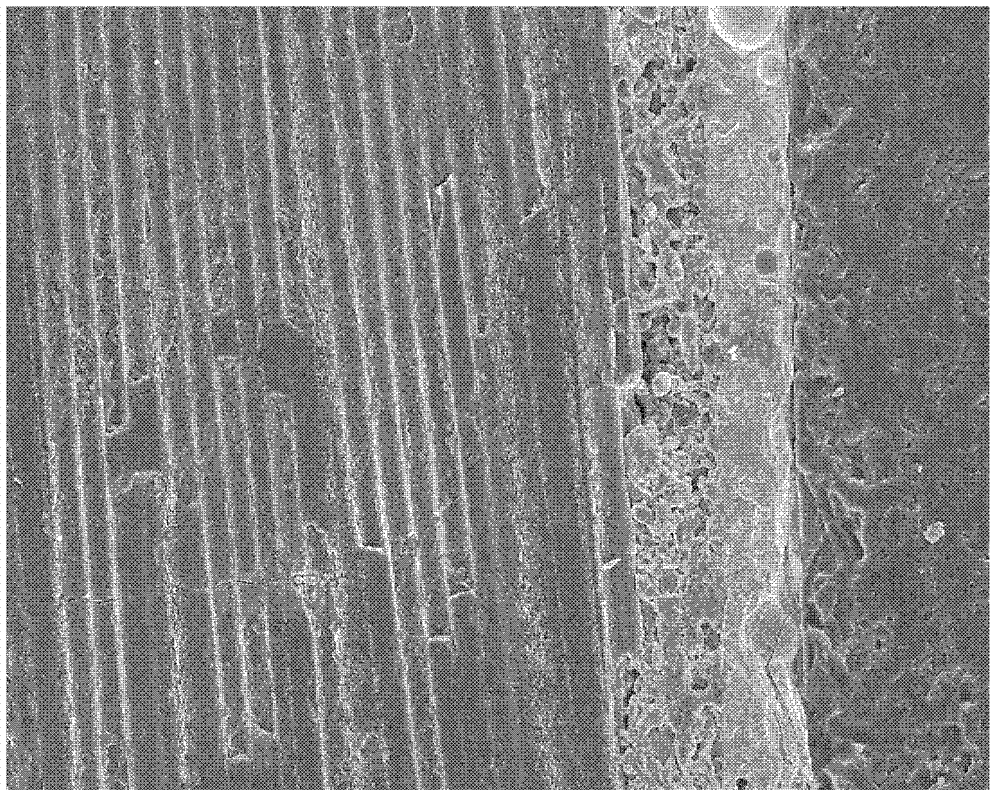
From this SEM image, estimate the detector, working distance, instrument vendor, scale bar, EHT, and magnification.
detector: SE(M)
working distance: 14.3 mm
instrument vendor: Hitachi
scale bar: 100000 nm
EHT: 10 kV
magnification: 0.5 K X